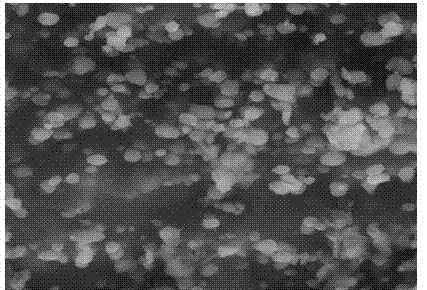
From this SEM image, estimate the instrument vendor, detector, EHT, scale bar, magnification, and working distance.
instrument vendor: Hitachi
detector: SE(M)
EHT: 15 kV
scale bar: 5000 nm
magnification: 10 K X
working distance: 12.5 mm